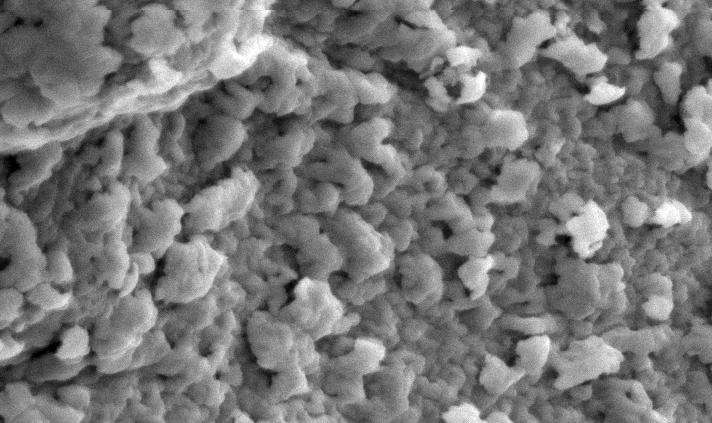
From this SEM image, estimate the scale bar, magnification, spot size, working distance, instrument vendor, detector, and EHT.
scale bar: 5000 nm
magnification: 5 K X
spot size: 3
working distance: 8.3 mm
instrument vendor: FEI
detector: SE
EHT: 10 kV